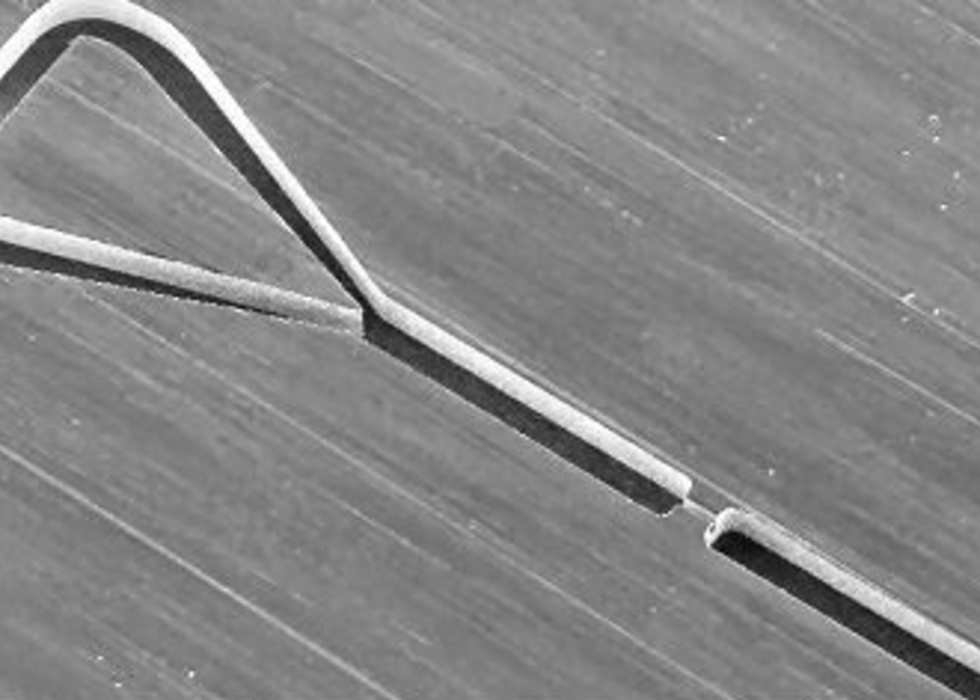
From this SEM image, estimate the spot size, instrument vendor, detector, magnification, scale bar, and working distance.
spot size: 3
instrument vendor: FEI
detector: SE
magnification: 0.1 K X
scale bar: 500000 nm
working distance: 11.9 mm